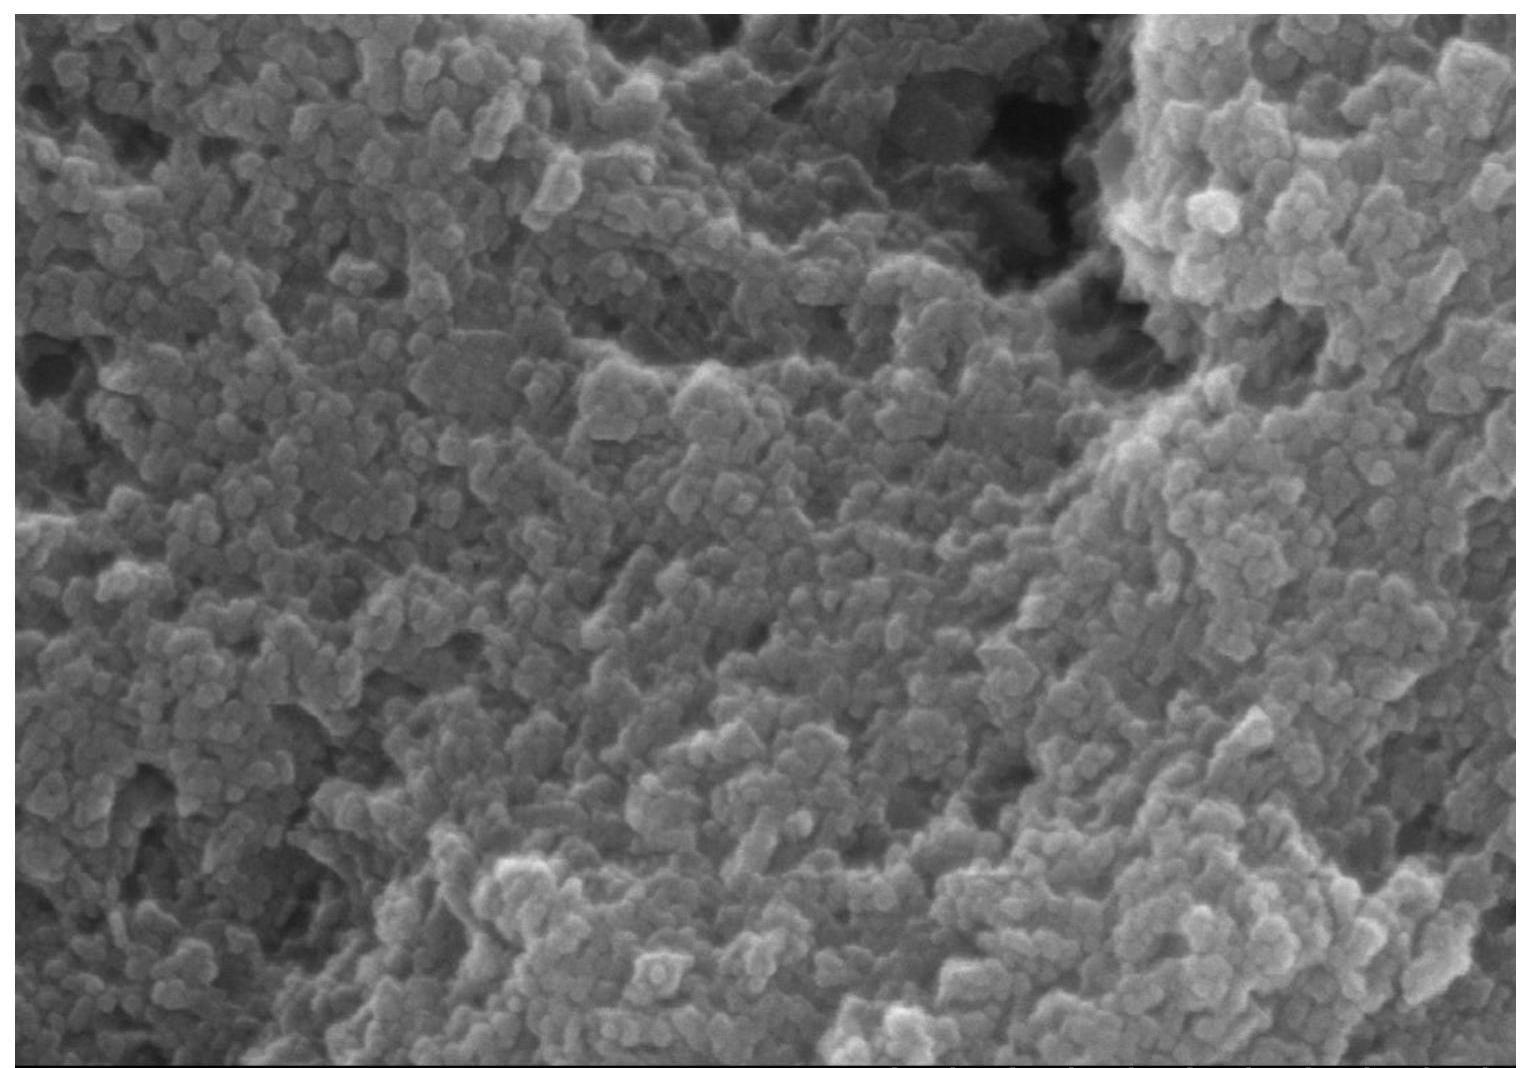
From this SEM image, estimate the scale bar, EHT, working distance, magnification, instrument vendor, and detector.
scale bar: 500 nm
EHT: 10 kV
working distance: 11.6 mm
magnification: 100 K X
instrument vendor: Hitachi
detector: SE(M)